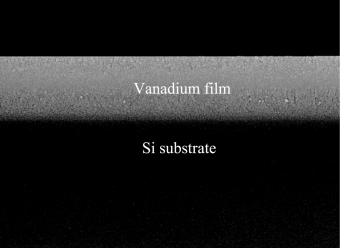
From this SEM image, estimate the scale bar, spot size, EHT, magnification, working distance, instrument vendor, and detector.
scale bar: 1000 nm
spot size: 5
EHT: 20 kV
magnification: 19.996 K X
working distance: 5.4 mm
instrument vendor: FEI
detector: TLD